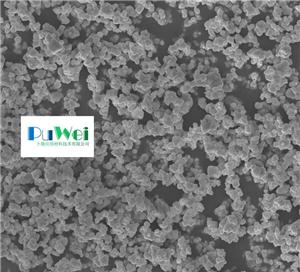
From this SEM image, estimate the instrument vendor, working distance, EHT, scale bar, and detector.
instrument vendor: Zeiss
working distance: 5.6 mm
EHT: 10 kV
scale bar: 1000 nm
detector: InLens